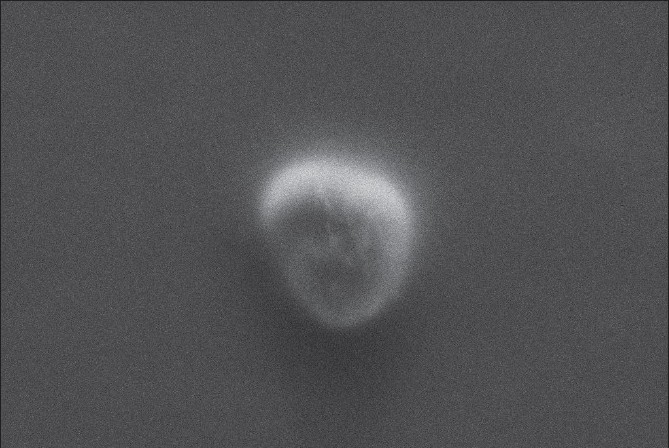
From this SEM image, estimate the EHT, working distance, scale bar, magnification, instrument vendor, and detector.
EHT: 15 kV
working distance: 25 mm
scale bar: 2000 nm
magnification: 2.44 K X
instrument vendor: Zeiss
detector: SE1